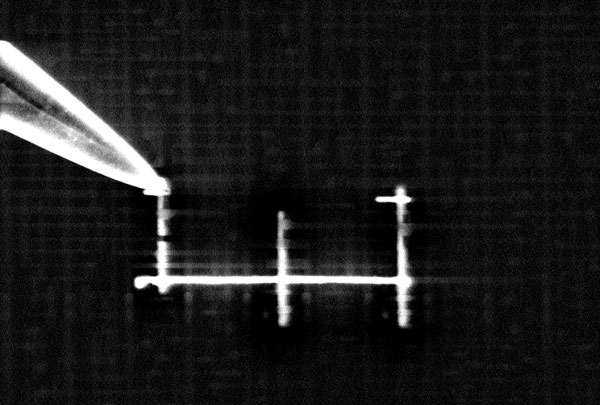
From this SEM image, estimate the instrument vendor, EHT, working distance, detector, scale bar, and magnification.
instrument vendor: Zeiss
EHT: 8 kV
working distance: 3.9 mm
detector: InLens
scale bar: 200 nm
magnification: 20 K X